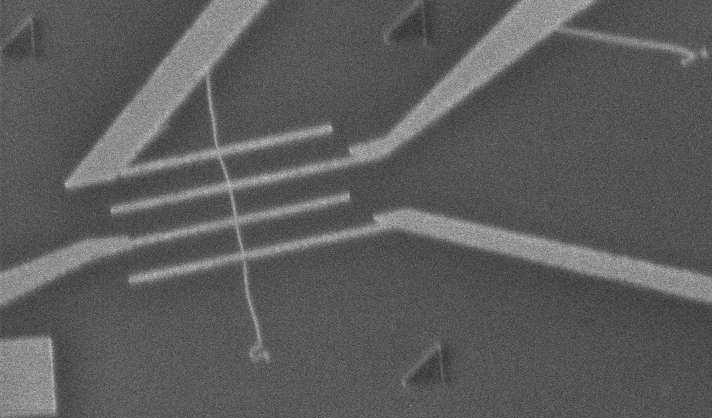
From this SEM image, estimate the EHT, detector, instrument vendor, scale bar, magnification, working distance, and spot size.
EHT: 1 kV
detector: TLD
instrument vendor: FEI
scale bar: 5000 nm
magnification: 6.917 K X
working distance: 5.8 mm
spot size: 3